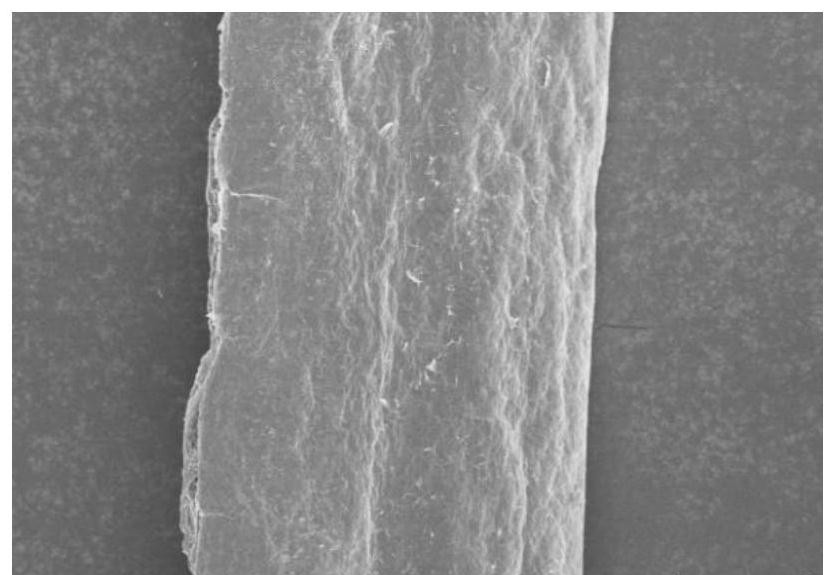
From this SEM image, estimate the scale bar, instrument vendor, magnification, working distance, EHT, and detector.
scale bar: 100000 nm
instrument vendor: Hitachi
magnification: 0.4 K X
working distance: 8.7 mm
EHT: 5 kV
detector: SE(UL)